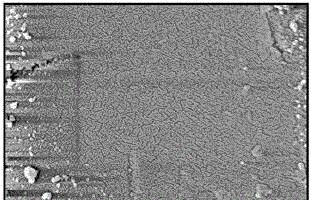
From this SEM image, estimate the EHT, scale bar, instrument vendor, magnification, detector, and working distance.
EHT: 3 kV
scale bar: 1000 nm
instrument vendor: Hitachi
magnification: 50 K X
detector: SE(M)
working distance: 2.8 mm